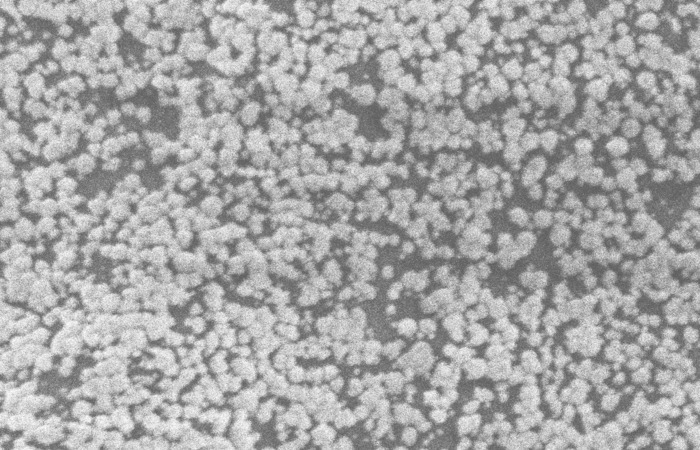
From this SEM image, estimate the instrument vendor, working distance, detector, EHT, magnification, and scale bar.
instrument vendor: Zeiss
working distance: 8.6 mm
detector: SE2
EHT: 5 kV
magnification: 32.49 K X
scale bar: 2000 nm